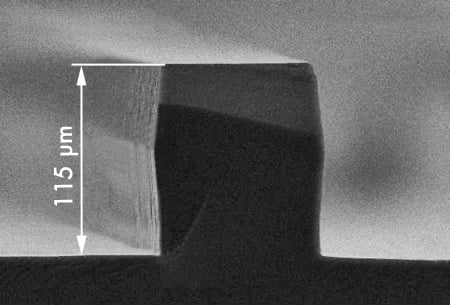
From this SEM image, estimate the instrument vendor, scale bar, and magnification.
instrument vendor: other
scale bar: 50000 nm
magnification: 0.45 K X